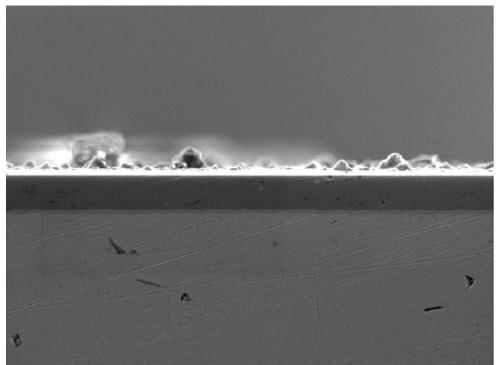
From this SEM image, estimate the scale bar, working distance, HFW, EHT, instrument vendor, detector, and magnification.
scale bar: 10000 nm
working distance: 19.51 mm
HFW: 55.4 µm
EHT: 5 kV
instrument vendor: Tescan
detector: SE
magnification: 5 K X